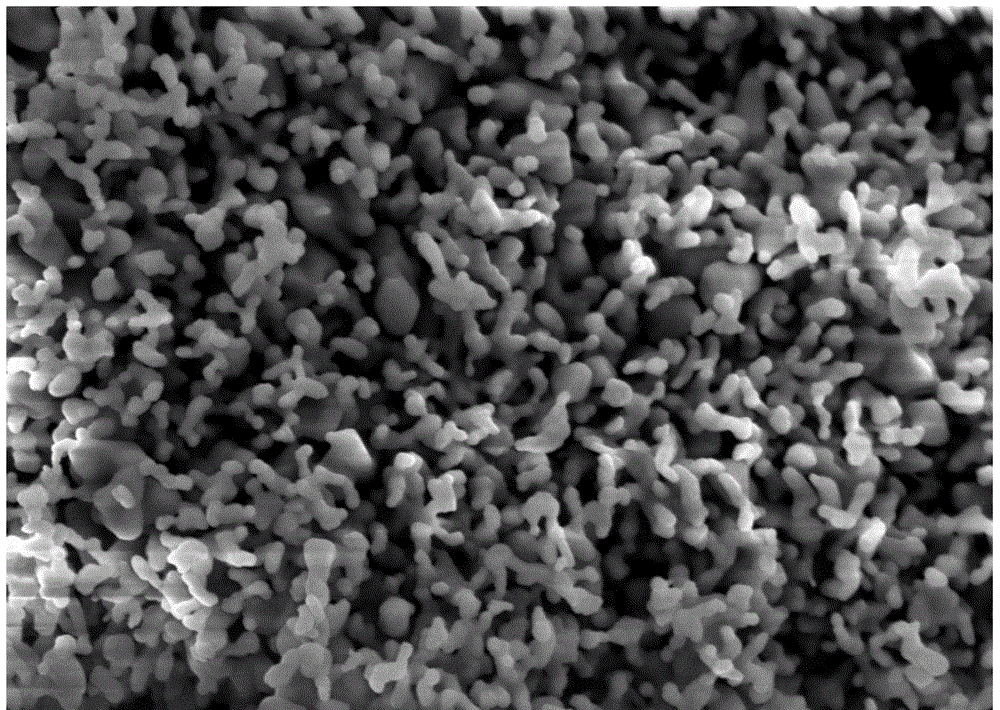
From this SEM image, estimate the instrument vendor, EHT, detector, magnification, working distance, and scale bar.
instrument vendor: Zeiss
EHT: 5 kV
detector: SE2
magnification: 20 K X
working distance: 6.3 mm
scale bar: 200 nm